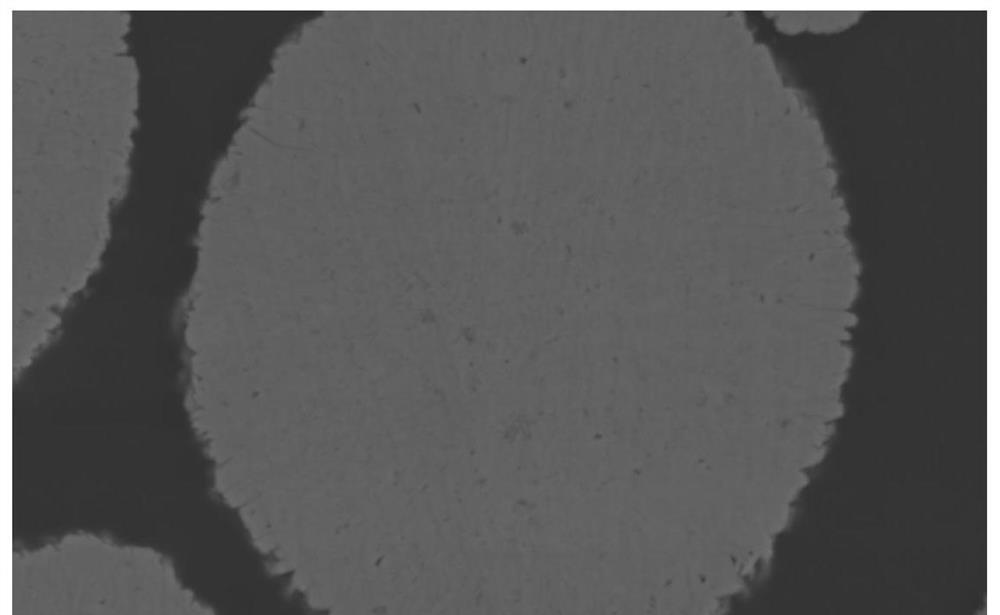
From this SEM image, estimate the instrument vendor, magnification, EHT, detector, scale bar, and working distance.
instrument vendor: Hitachi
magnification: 10 K X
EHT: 5 kV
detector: SE(M,LA100)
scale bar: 5000 nm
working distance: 7.8 mm